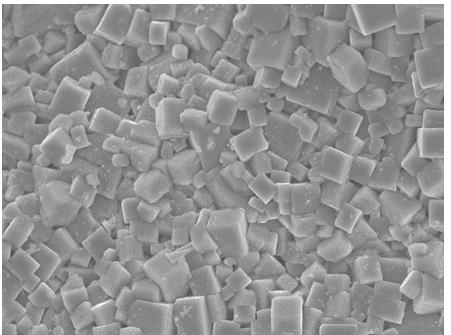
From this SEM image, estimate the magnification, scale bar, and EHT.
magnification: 3 K X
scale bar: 10000 nm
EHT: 15 kV